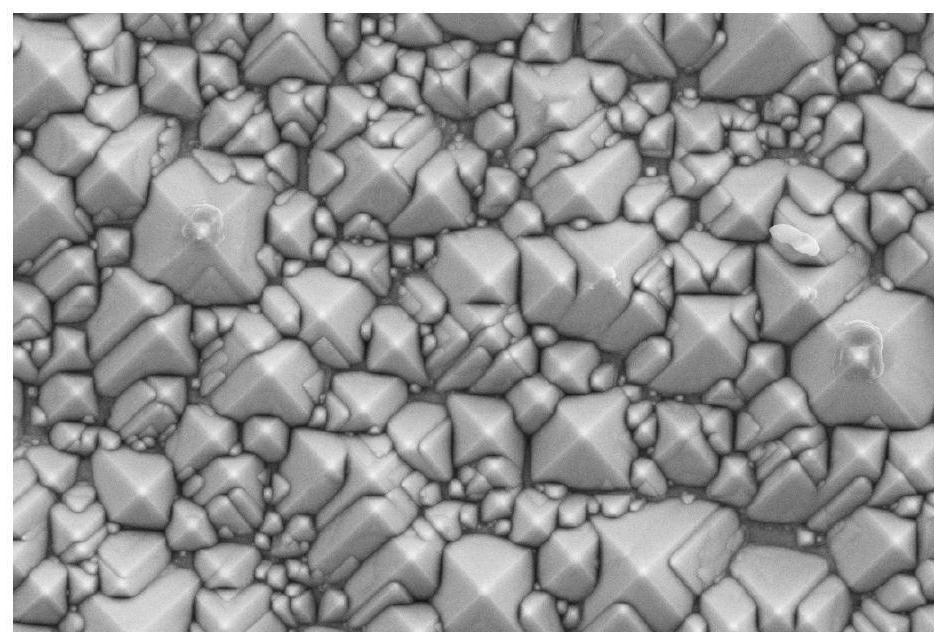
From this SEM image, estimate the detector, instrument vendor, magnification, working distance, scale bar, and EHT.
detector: SE2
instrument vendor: Zeiss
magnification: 5 K X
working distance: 8.6 mm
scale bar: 1000 nm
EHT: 5 kV